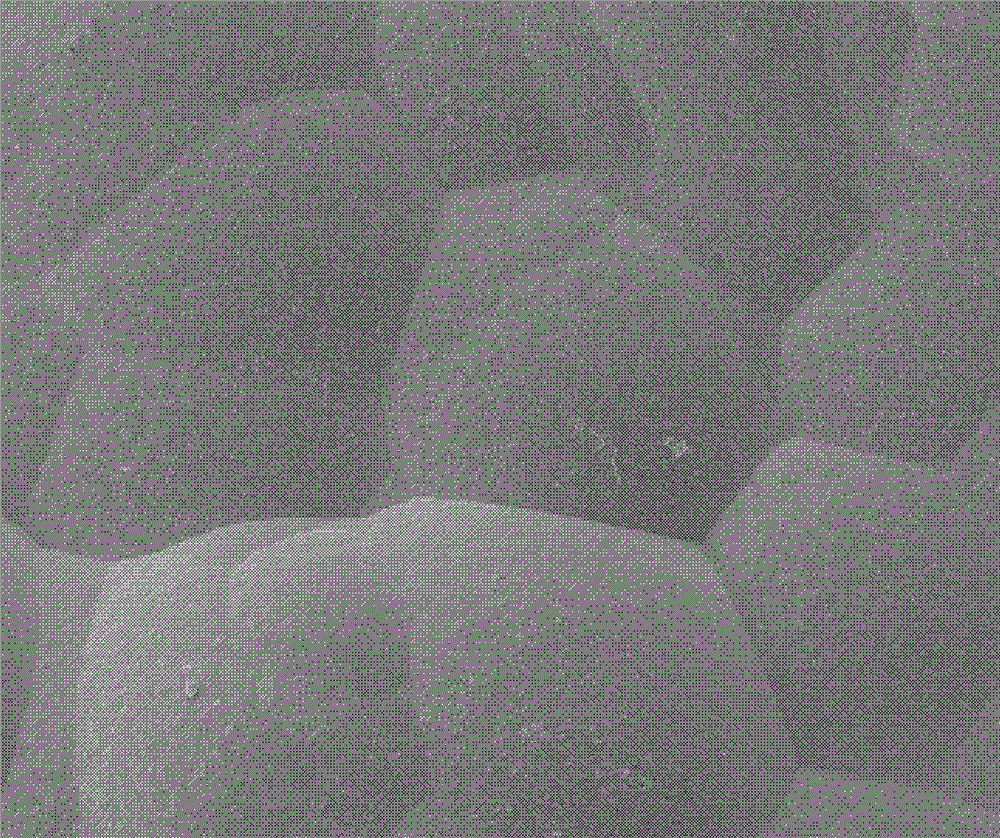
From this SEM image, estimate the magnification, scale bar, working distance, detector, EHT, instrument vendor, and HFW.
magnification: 2 K X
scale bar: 30000 nm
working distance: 4.6 mm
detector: ETD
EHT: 5 kV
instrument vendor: FEI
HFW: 120 µm